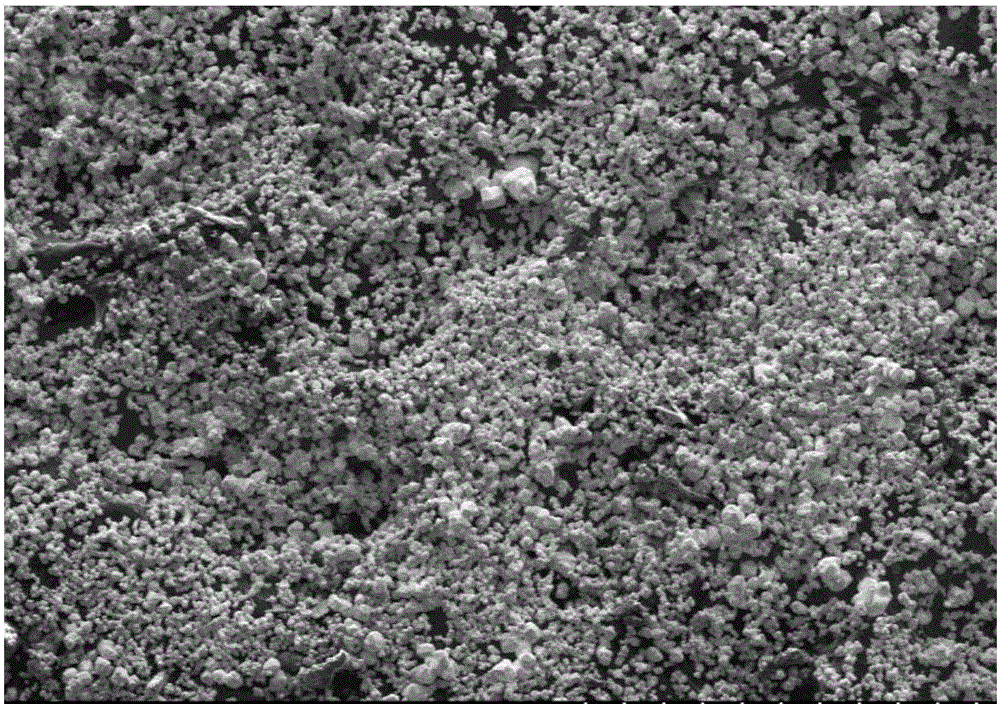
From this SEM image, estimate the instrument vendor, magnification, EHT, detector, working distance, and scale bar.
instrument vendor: Hitachi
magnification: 0.1 K X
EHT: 10 kV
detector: SE(M)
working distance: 10.7 mm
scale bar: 500000 nm